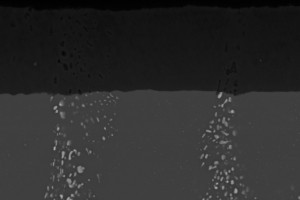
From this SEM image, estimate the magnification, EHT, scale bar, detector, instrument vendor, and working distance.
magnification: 0.9 K X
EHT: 20 kV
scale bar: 10000 nm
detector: BSD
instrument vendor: Zeiss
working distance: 24 mm